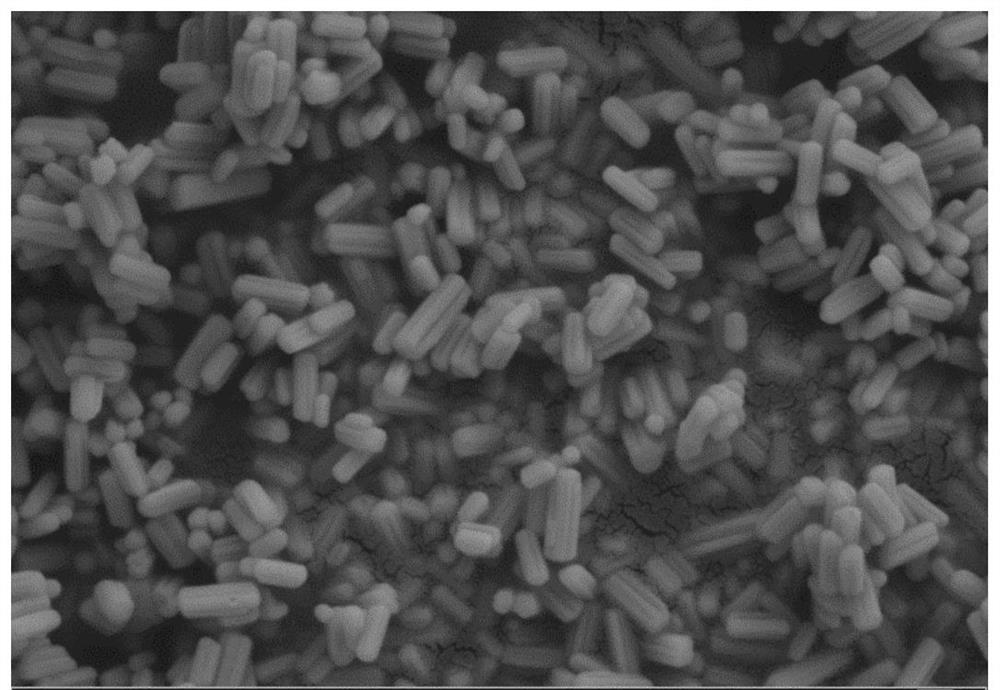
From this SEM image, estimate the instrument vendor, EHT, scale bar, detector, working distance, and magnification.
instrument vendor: Zeiss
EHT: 5 kV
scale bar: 300 nm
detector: SE2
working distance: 9.4 mm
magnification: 27.66 K X